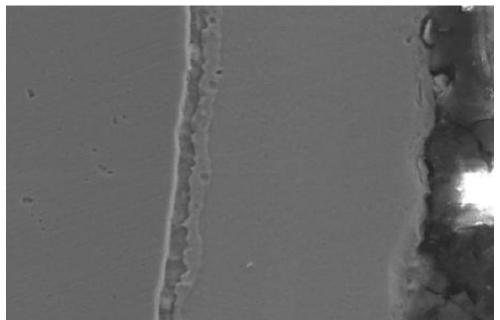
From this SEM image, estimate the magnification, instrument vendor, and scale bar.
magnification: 1 K X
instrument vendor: JEOL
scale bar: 10000 nm